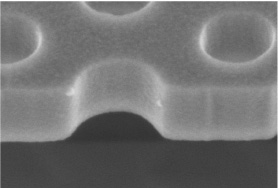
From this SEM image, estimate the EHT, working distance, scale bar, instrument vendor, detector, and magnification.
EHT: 2 kV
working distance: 5 mm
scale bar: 300 nm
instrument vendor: Hitachi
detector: SE(M)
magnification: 150 K X